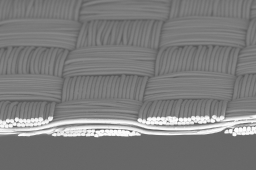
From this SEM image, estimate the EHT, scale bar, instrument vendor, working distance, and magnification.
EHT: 15 kV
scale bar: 100000 nm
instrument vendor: JEOL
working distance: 20.1 mm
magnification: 0.3 K X